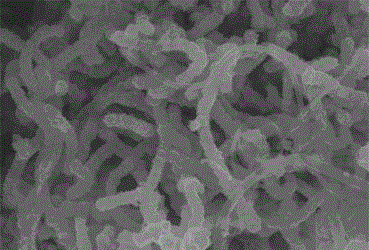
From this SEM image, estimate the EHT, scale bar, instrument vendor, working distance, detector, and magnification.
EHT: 10 kV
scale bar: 1000 nm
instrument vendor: Hitachi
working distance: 8.4 mm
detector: SE(M)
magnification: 30 K X